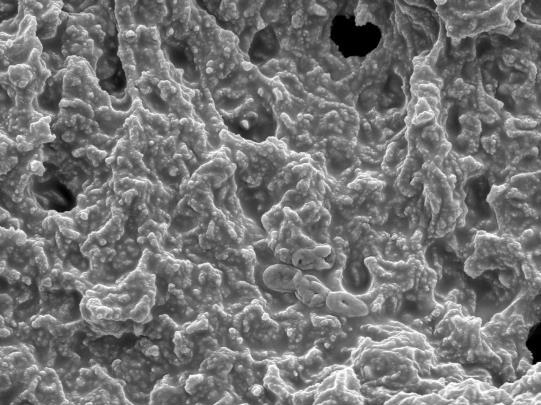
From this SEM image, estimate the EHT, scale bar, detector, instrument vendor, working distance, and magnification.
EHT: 10 kV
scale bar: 1000 nm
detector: SEI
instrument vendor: JEOL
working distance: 6.3 mm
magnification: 8 K X